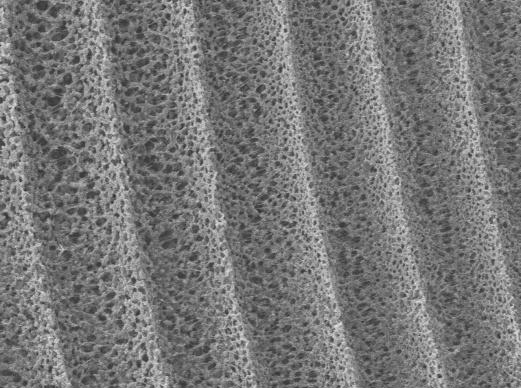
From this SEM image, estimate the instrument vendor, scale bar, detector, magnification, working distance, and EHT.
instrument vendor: JEOL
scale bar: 10000 nm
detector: LEI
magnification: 0.8 K X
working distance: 8 mm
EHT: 10 kV